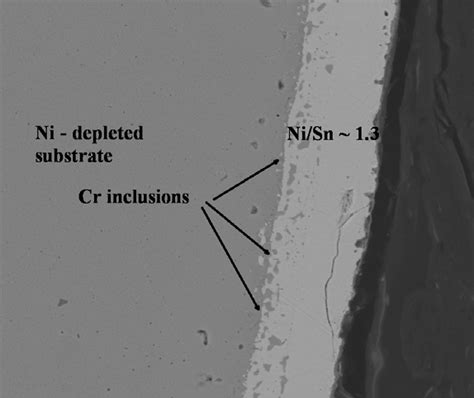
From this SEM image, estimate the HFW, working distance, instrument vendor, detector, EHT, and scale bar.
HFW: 64 µm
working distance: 10 mm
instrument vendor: FEI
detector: BSE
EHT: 15 kV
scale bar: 20000 nm